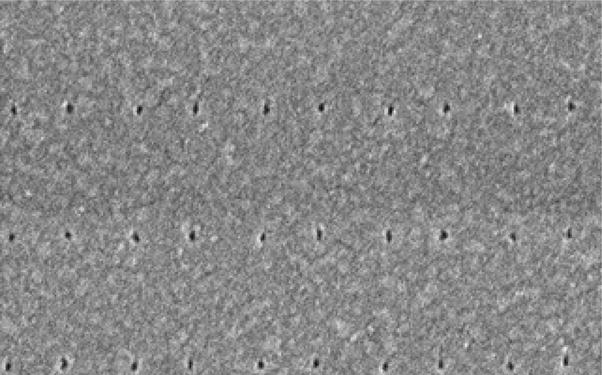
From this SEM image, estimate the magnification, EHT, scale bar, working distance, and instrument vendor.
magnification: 130 K X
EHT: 10 kV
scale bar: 400 nm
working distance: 7.6 mm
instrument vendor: Hitachi